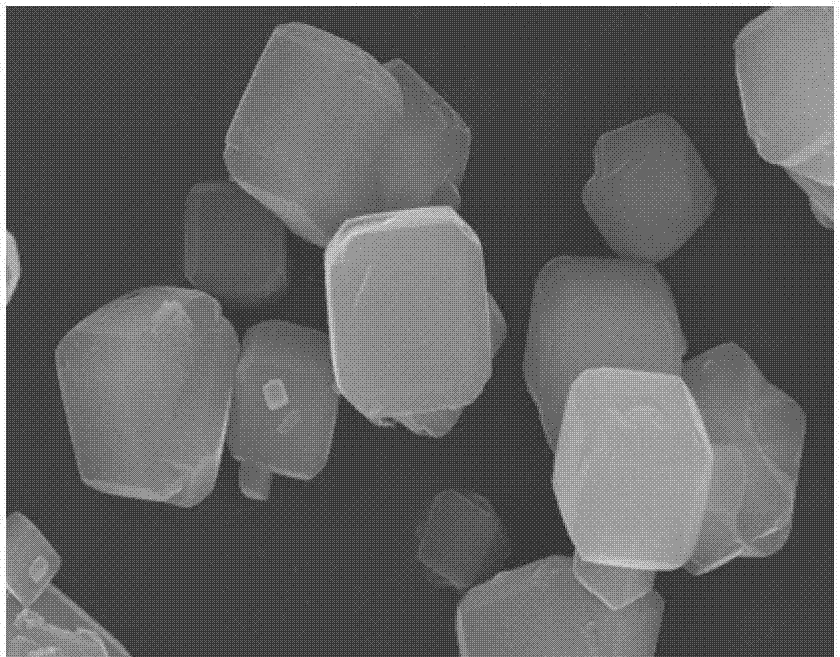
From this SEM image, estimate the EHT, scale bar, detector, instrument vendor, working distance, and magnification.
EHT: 15 kV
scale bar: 5000 nm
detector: SE(M)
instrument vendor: Hitachi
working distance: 15.9 mm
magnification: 10 K X